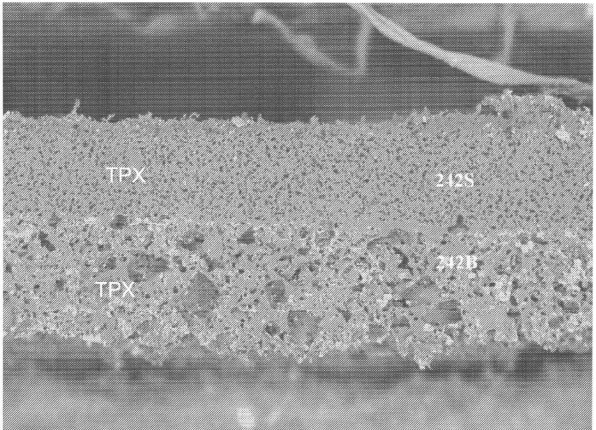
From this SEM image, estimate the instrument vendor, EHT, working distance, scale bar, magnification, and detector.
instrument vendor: JEOL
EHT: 10 kV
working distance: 16 mm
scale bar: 100000 nm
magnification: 0.2 K X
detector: COMPO